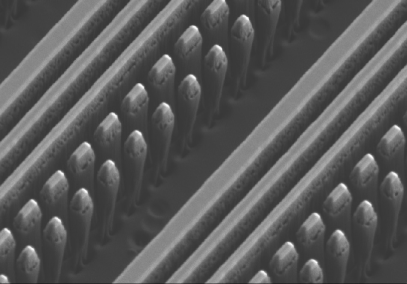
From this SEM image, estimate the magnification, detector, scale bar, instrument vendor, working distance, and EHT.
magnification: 22 K X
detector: SE(L)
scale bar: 2000 nm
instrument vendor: Hitachi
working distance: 9 mm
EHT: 2 kV